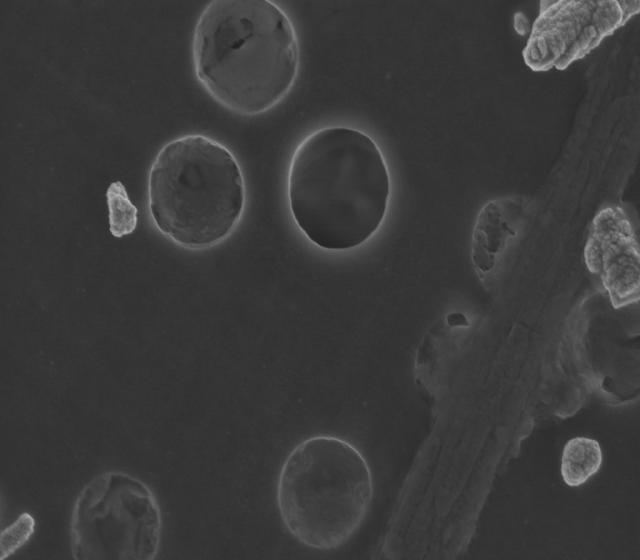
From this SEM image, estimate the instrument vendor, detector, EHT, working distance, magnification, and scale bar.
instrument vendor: Zeiss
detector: InLens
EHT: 3 kV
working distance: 3 mm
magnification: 65 K X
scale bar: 200 nm